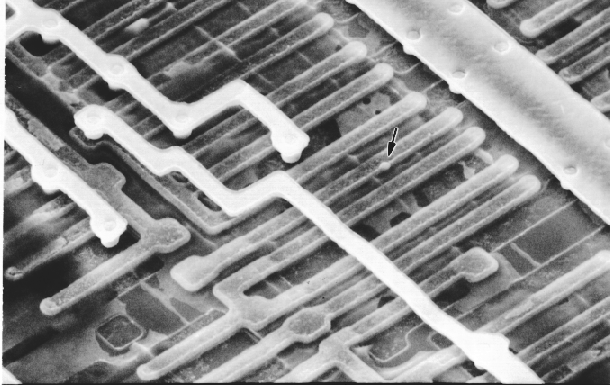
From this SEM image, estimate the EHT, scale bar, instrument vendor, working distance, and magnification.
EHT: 15 kV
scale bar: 10000 nm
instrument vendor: JEOL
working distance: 20 mm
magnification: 3.5 K X